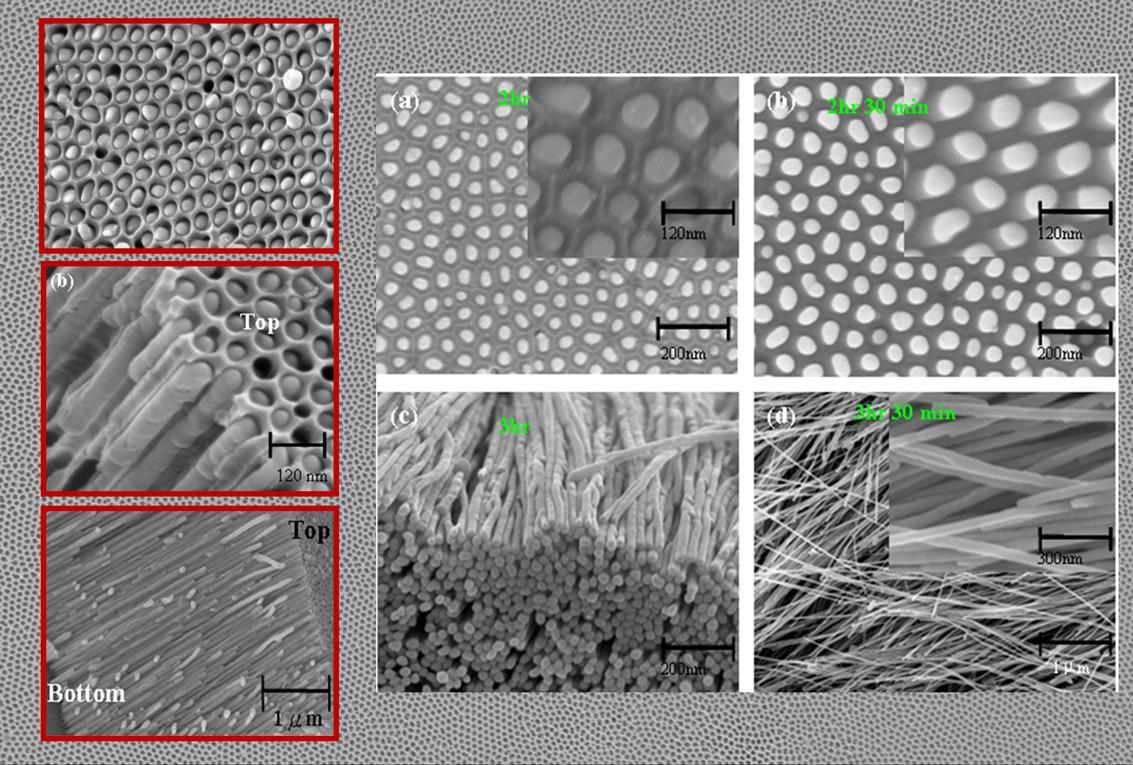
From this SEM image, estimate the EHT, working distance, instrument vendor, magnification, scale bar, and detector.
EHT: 10 kV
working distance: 1 mm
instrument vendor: Zeiss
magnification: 8 K X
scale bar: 1000 nm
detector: InLens